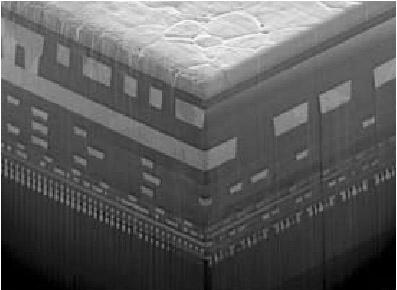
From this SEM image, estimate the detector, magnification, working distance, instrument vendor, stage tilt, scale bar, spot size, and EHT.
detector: TLD-S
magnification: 15 K X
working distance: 4.855 mm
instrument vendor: FEI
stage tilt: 59°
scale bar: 5000 nm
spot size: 4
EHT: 5 kV